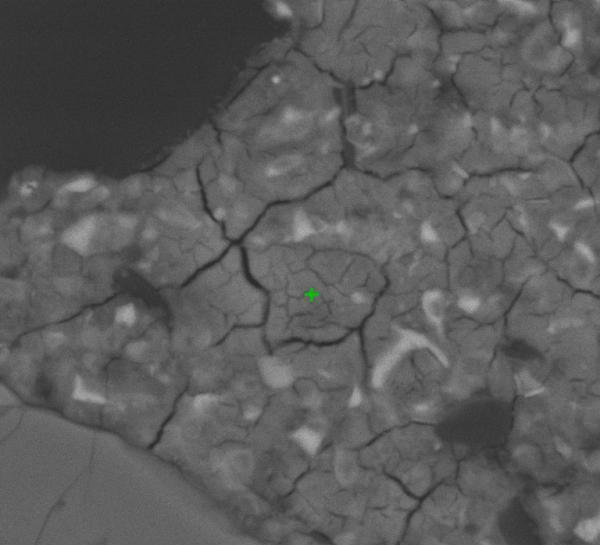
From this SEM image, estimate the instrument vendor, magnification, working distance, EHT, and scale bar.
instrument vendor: other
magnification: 2 K X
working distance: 16.6 mm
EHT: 30 kV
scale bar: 7000 nm